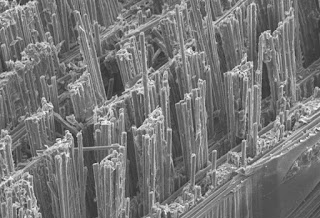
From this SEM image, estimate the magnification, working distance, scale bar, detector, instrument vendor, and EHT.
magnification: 0.2 K X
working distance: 63.2 mm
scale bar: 200000 nm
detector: SE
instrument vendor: Hitachi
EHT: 15 kV